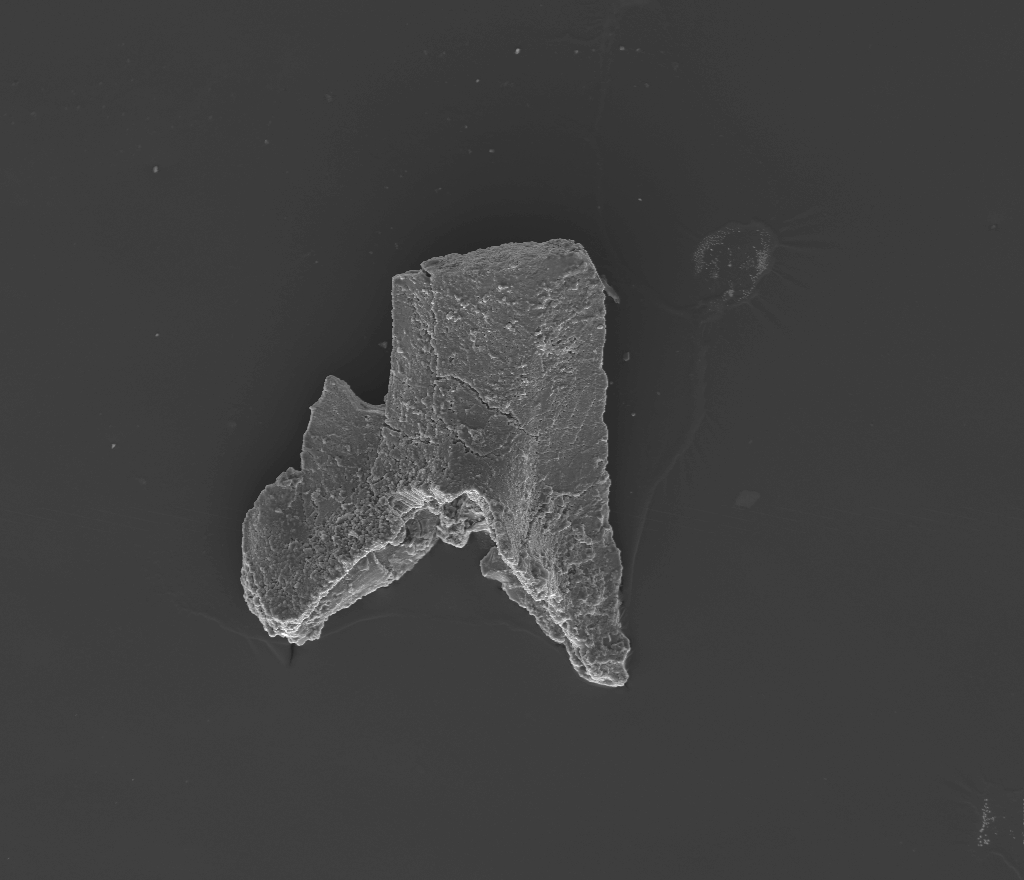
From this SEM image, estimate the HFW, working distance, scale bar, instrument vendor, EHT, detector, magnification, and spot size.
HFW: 870 µm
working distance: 10 mm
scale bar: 200000 nm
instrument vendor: FEI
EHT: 20 kV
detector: ETD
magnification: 0.31 K X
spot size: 5.5